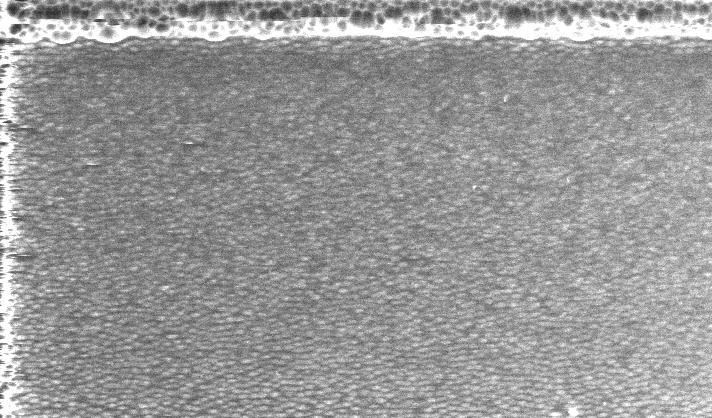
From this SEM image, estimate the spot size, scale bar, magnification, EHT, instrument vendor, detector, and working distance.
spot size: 5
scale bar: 20000 nm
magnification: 1 K X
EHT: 10 kV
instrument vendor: FEI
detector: GSE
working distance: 6.3 mm